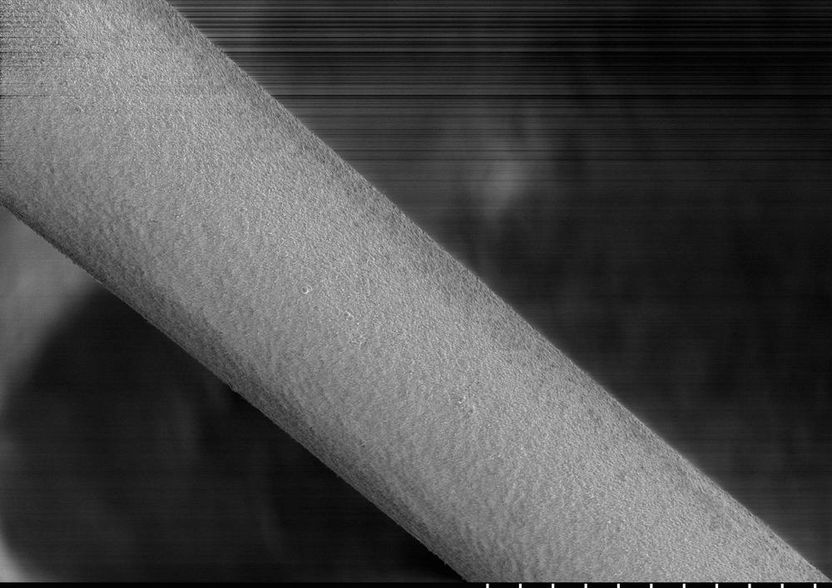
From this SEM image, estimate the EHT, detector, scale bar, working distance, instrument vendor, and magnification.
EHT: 1 kV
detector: SE(M)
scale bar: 50000 nm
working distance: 10.7 mm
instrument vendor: Hitachi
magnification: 1 K X